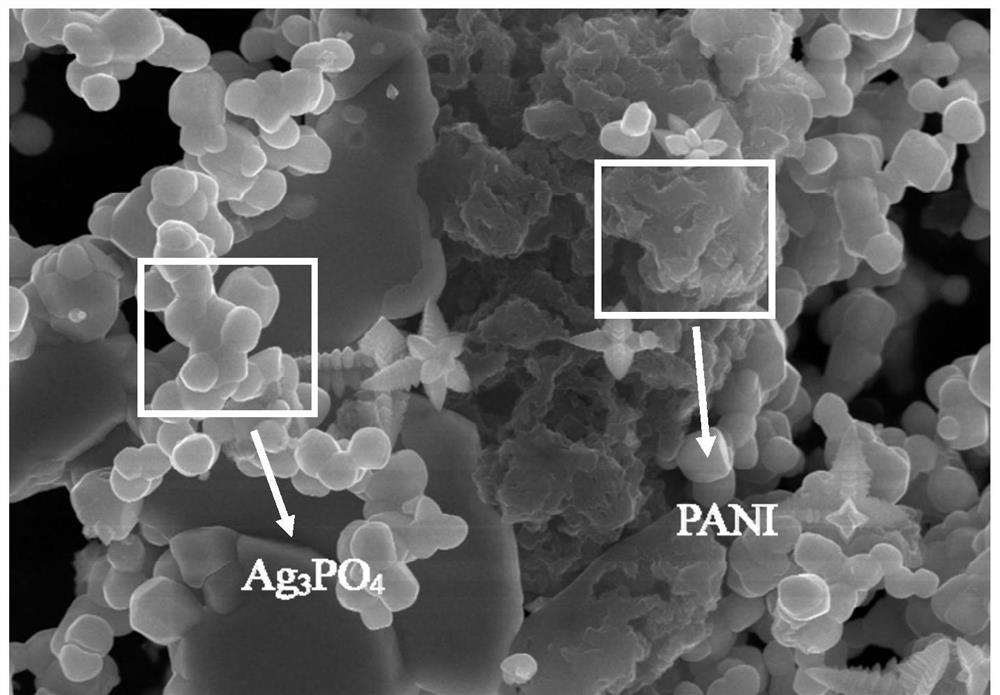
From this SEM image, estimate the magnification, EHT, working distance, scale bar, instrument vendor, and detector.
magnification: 10 K X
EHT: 15 kV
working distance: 10.1 mm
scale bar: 5000 nm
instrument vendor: Hitachi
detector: SE(UL)